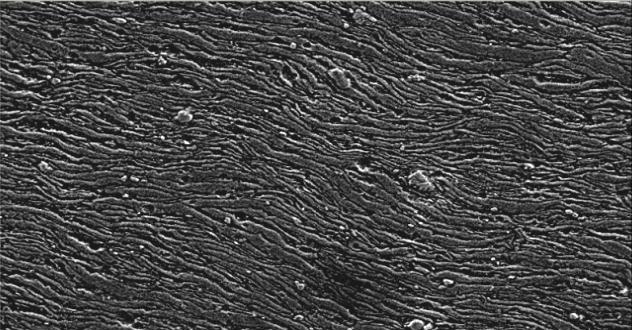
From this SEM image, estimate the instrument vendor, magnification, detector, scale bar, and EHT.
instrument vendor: JEOL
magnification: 0.5 K X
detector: SEI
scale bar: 50000 nm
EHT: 15 kV